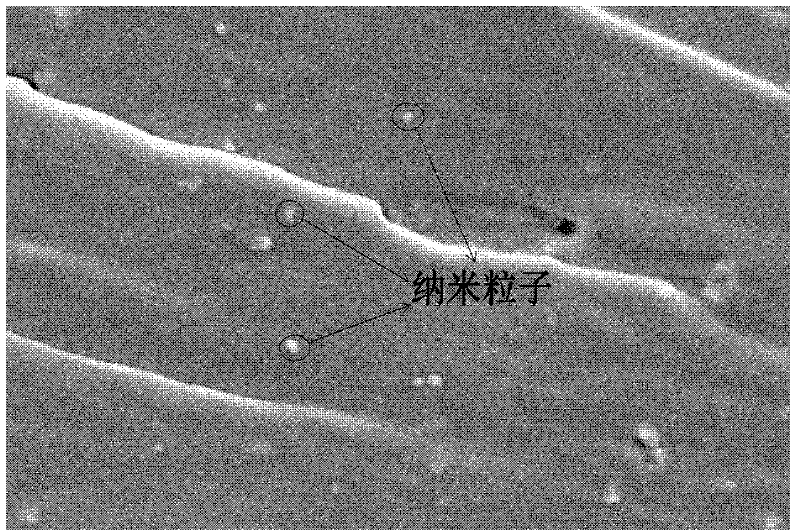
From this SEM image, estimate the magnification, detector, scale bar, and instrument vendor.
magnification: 10 K X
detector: SEI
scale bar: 1000 nm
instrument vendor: JEOL